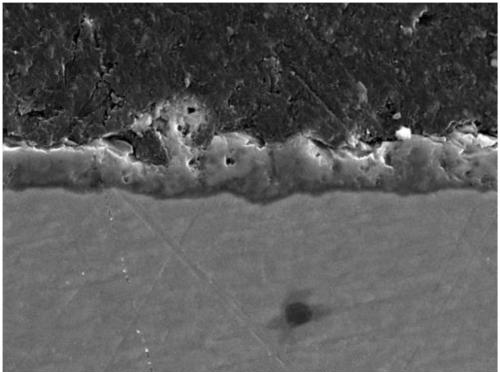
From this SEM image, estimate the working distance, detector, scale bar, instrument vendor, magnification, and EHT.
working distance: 9.14 mm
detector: SE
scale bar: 20000 nm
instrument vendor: Tescan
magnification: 2 K X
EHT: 30 kV